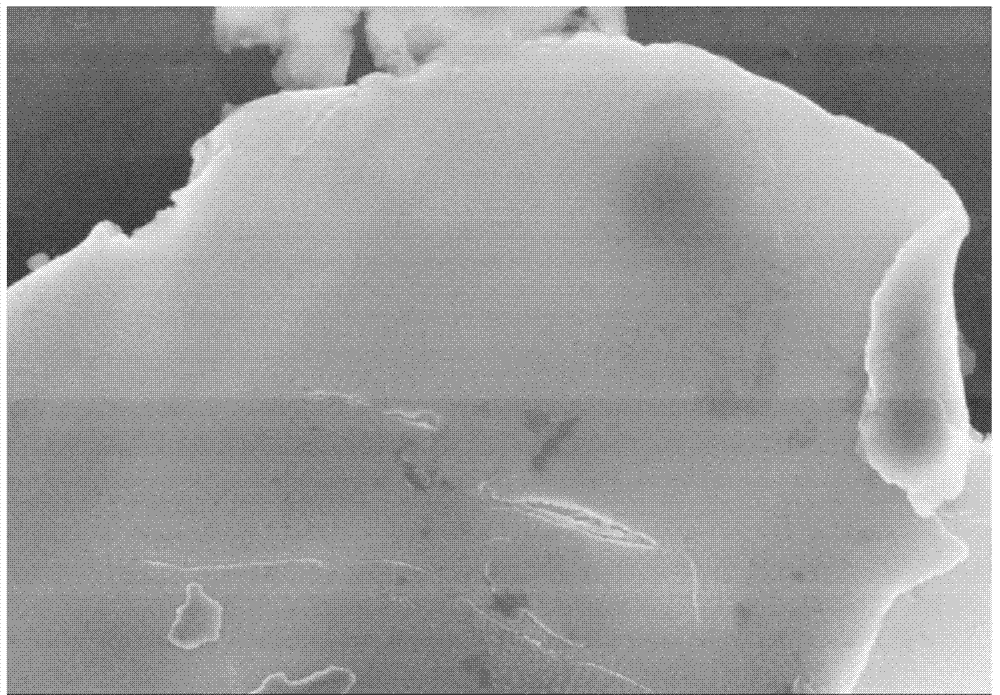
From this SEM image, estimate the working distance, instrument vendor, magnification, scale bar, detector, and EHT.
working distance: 9.5 mm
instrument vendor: Hitachi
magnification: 25 K X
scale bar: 2000 nm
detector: SE(M)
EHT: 5 kV